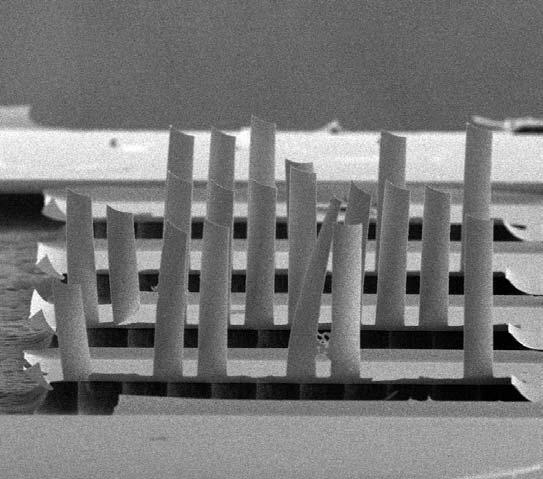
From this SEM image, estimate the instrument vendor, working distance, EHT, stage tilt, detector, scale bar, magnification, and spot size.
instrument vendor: FEI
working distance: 8.65 mm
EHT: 25 kV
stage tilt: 40°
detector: SED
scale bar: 100000 nm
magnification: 0.35 K X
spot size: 3